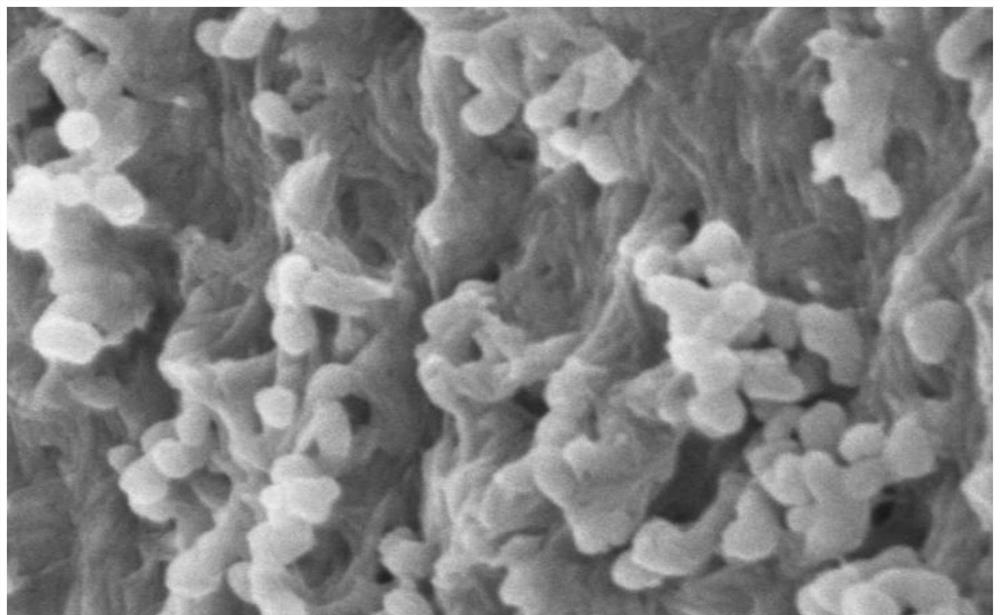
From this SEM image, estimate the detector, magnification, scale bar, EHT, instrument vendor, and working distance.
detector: SE(UL)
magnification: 80 K X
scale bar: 500 nm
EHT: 3 kV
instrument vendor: Hitachi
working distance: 8 mm